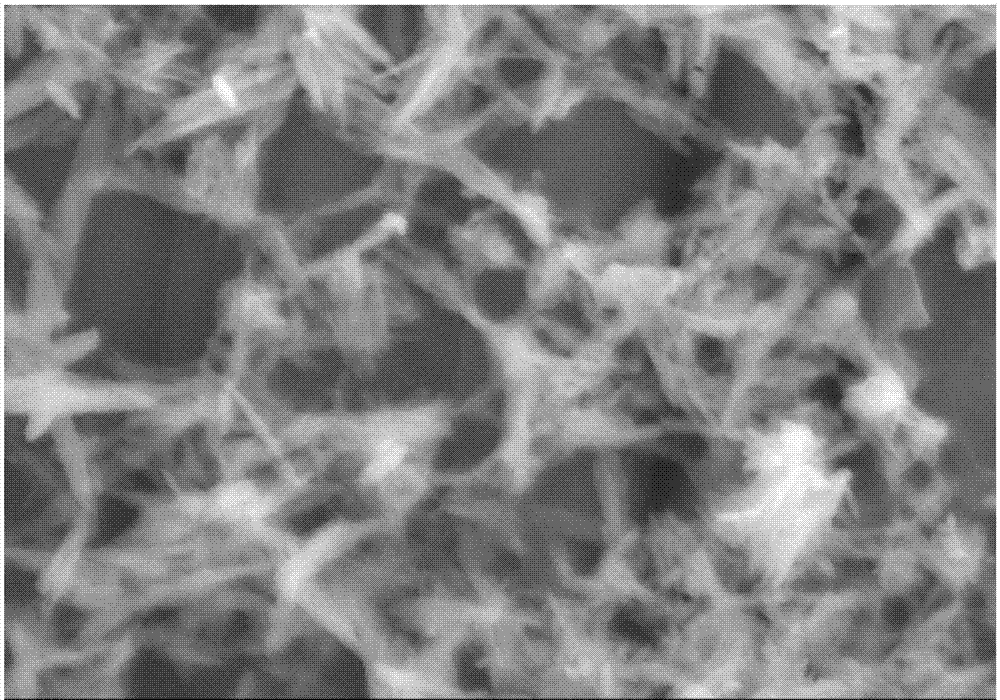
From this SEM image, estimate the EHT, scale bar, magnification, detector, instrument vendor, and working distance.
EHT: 5 kV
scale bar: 500 nm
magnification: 100 K X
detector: SE(U,LA0)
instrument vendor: Hitachi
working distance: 3.3 mm